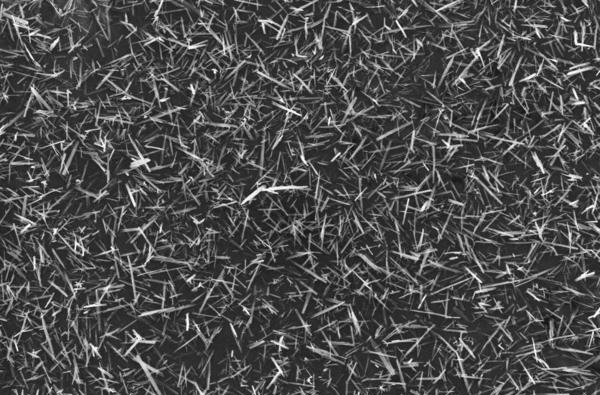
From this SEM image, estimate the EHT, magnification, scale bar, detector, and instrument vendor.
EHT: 20 kV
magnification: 0.2 K X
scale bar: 100000 nm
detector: SEI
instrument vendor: JEOL